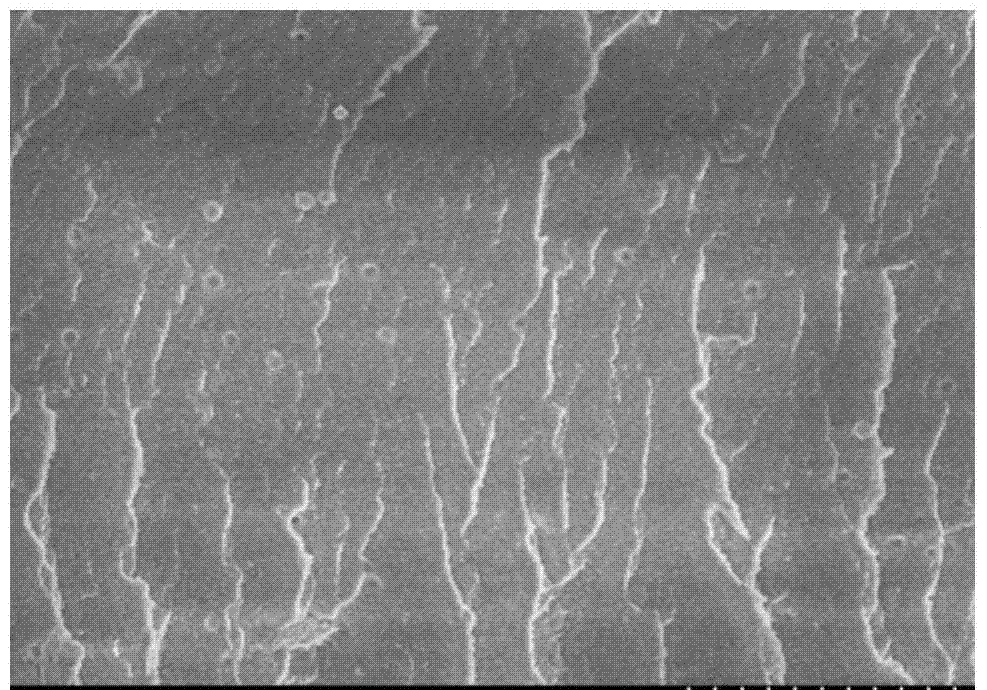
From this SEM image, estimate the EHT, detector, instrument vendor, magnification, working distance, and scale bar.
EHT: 3 kV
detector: SE(M)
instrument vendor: Hitachi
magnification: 7 K X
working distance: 8 mm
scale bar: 5000 nm